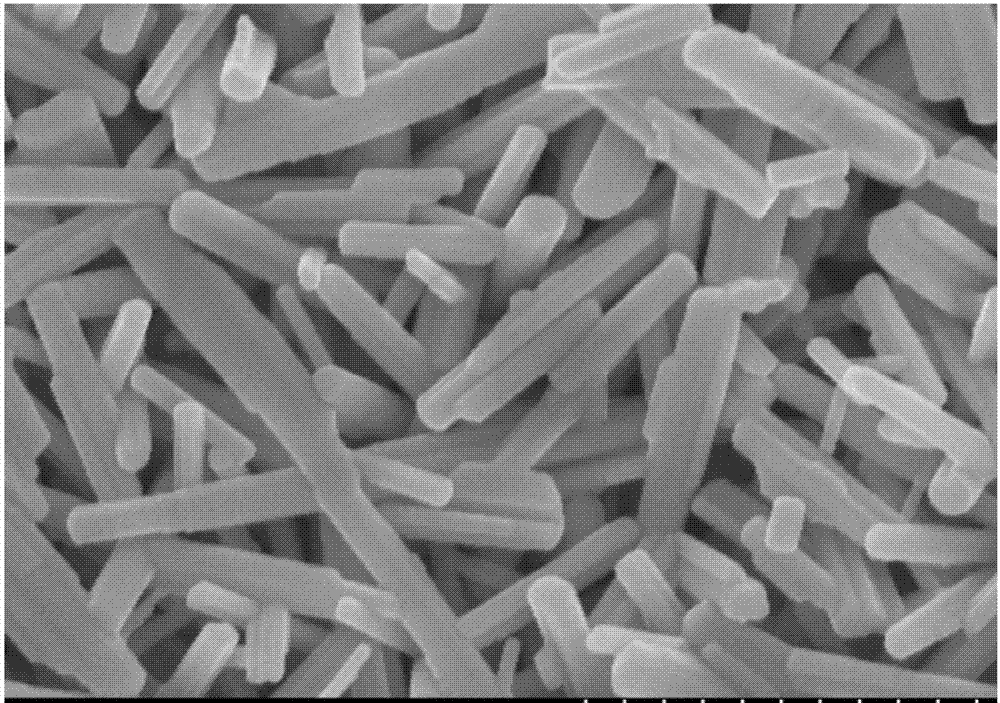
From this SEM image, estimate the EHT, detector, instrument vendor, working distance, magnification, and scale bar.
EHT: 5 kV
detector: SE(U)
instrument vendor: Hitachi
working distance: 6.2 mm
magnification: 50 K X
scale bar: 1000 nm